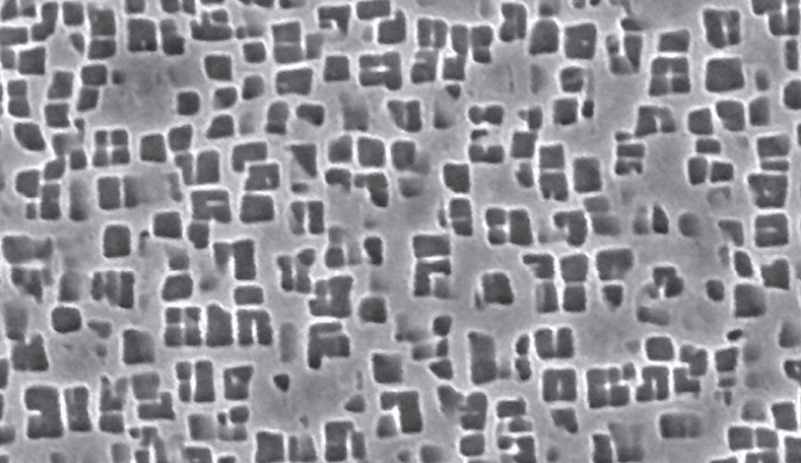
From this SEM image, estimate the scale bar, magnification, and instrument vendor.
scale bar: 10000 nm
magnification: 5 K X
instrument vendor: Hitachi